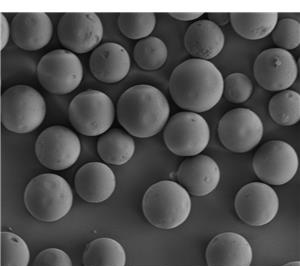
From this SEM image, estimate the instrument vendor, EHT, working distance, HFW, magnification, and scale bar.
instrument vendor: FEI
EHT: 3 kV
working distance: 4.9 mm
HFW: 533 µm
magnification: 0.28 K X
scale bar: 100000 nm